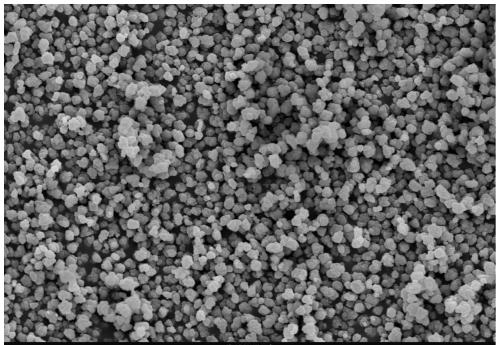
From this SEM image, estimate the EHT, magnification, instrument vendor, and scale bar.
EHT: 25 kV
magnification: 1 K X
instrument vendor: other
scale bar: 10000 nm